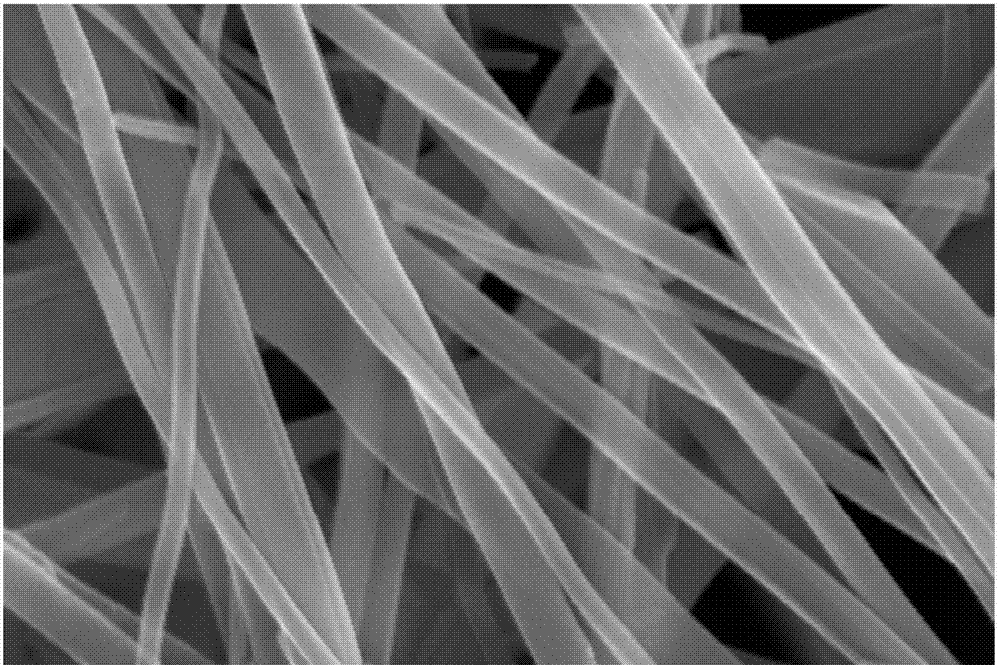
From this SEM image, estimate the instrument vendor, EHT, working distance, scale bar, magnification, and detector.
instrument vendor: Zeiss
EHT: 15 kV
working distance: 11.1 mm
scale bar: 200 nm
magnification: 30 K X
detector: InLens